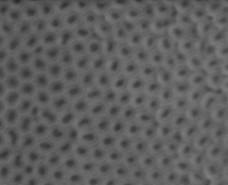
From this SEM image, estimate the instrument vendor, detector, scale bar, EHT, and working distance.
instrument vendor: FEI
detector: LFD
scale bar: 500 nm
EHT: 10 kV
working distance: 11.1 mm